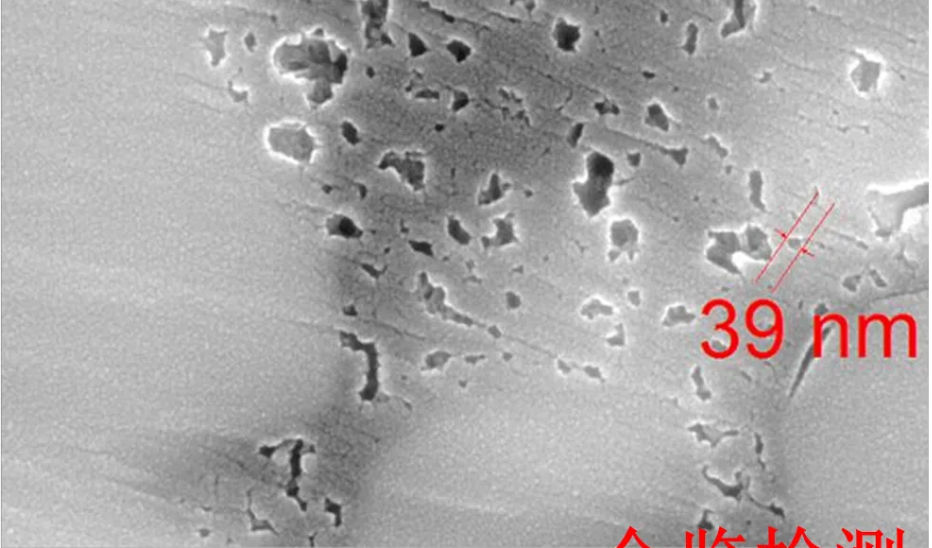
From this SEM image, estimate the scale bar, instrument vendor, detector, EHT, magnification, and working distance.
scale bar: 500 nm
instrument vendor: Tescan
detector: InBeam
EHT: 20 kV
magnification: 100 K X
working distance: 6.03 mm